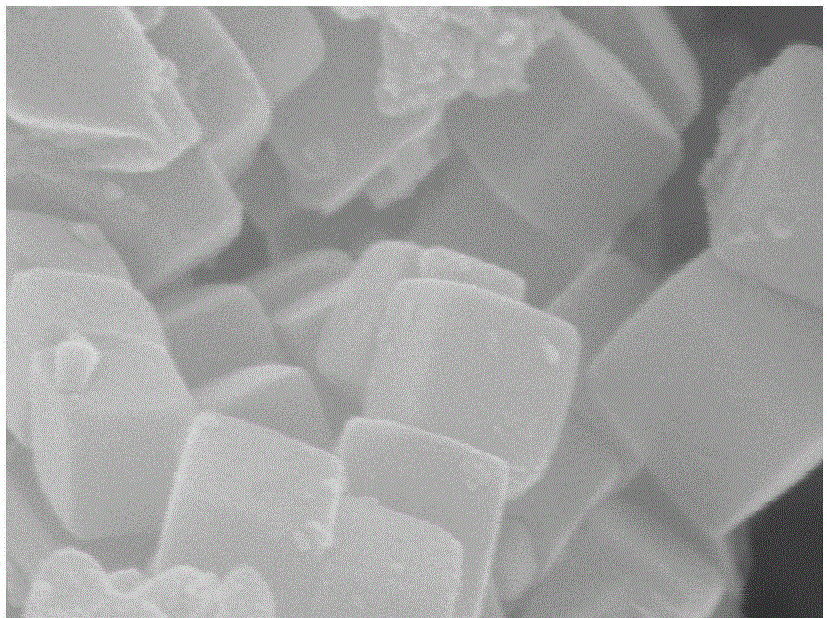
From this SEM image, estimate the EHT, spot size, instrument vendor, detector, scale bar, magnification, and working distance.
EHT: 10 kV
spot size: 3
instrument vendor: FEI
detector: TLD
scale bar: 200 nm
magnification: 100 K X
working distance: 6.6 mm